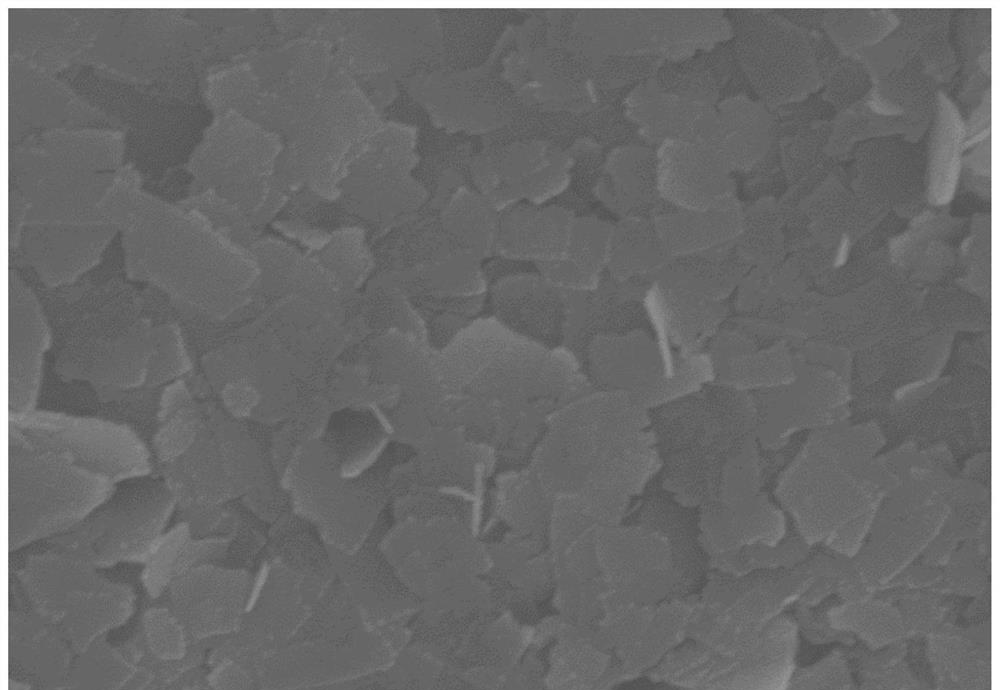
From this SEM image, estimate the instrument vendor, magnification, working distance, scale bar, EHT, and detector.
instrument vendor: Hitachi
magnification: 50 K X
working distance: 9 mm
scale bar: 1000 nm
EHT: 5 kV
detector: SE(M)